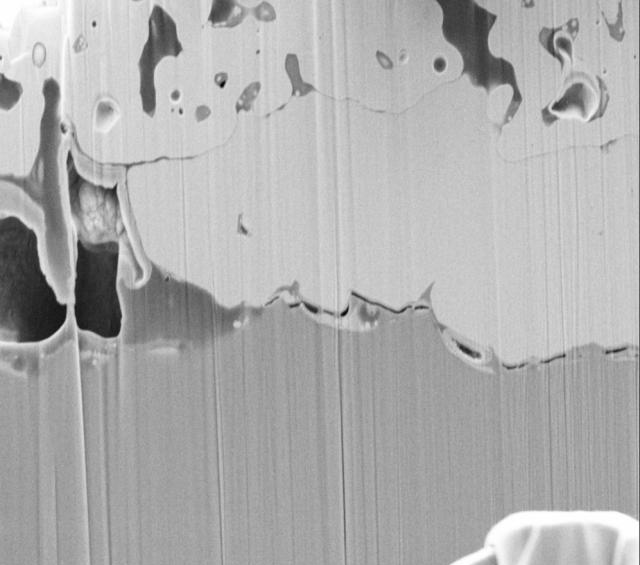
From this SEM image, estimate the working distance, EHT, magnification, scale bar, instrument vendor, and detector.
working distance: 4.7 mm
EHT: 4 kV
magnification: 5 K X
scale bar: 2000 nm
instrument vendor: Zeiss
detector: SE2